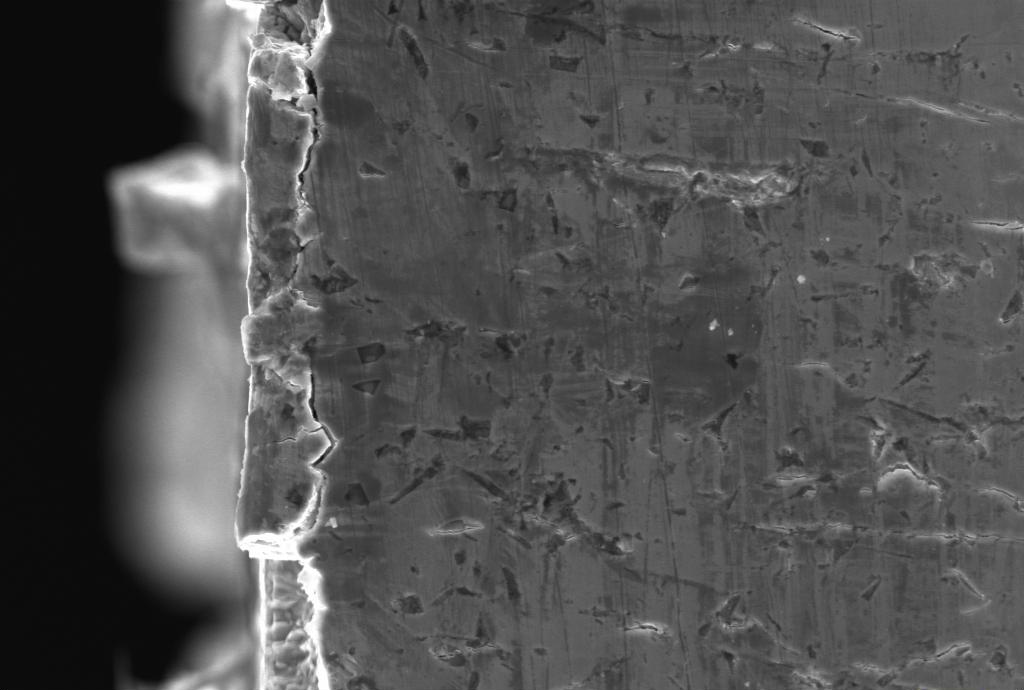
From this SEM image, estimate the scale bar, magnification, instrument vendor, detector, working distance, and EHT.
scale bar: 10000 nm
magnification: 2 K X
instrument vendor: Zeiss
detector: SE1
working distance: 30 mm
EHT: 20 kV